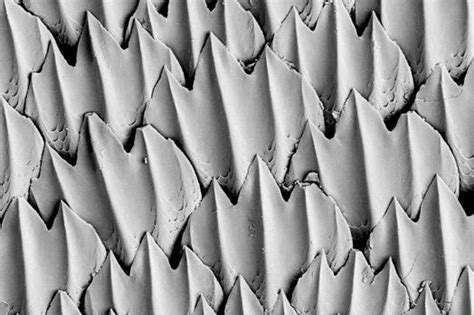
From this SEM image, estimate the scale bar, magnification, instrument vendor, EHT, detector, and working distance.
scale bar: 30000 nm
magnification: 0.484 K X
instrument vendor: Zeiss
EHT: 20 kV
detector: RBSD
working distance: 33 mm